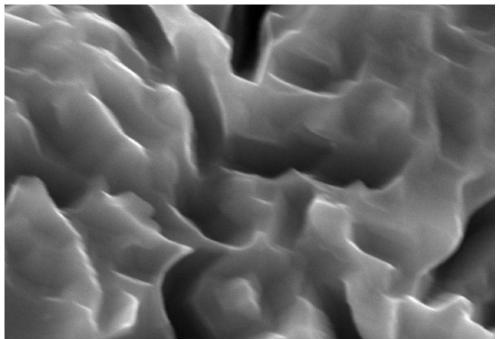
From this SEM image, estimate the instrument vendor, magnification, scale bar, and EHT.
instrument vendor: JEOL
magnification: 5 K X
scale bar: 5000 nm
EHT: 30 kV